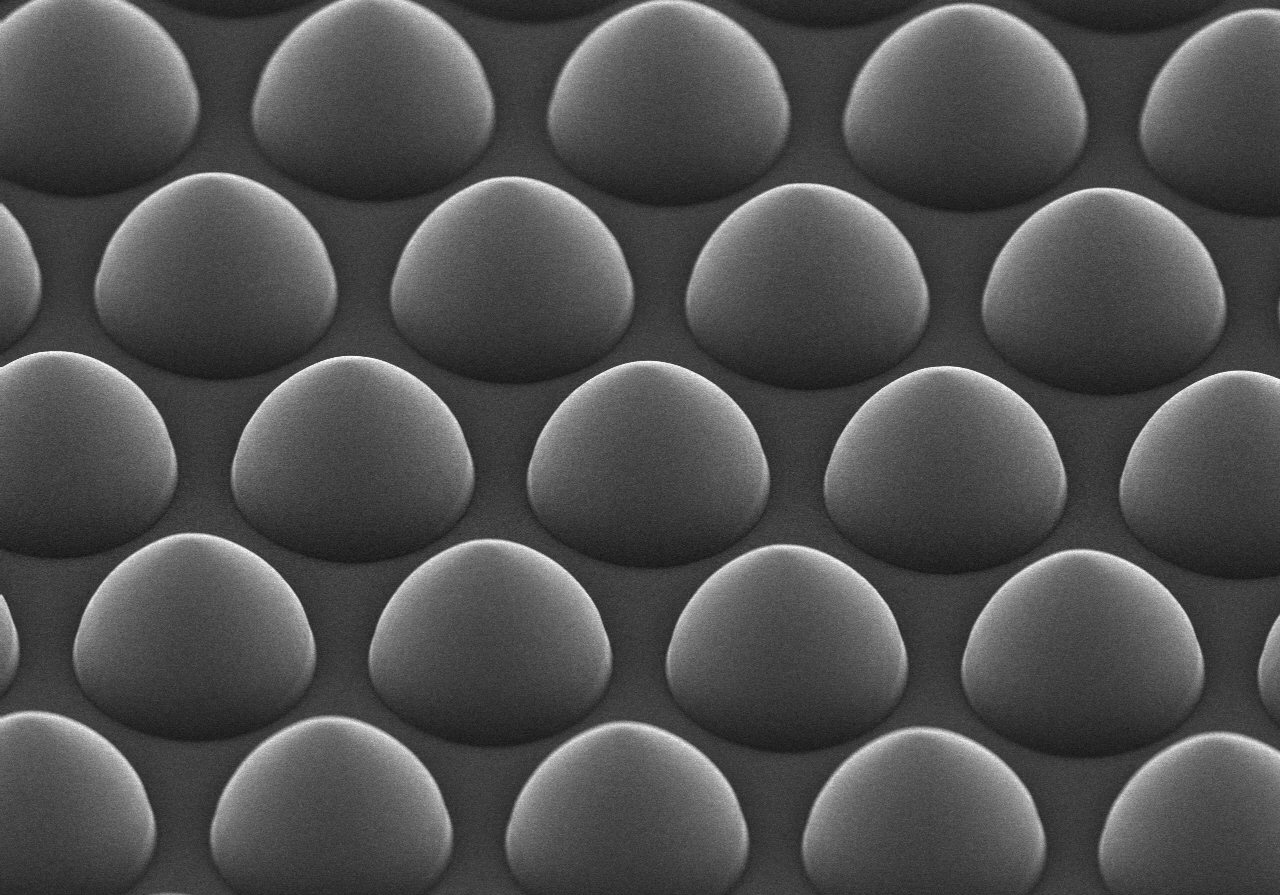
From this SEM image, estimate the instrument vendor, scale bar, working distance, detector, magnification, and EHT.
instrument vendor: Hitachi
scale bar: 5000 nm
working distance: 6.2 mm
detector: SE(U)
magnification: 10 K X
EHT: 15 kV